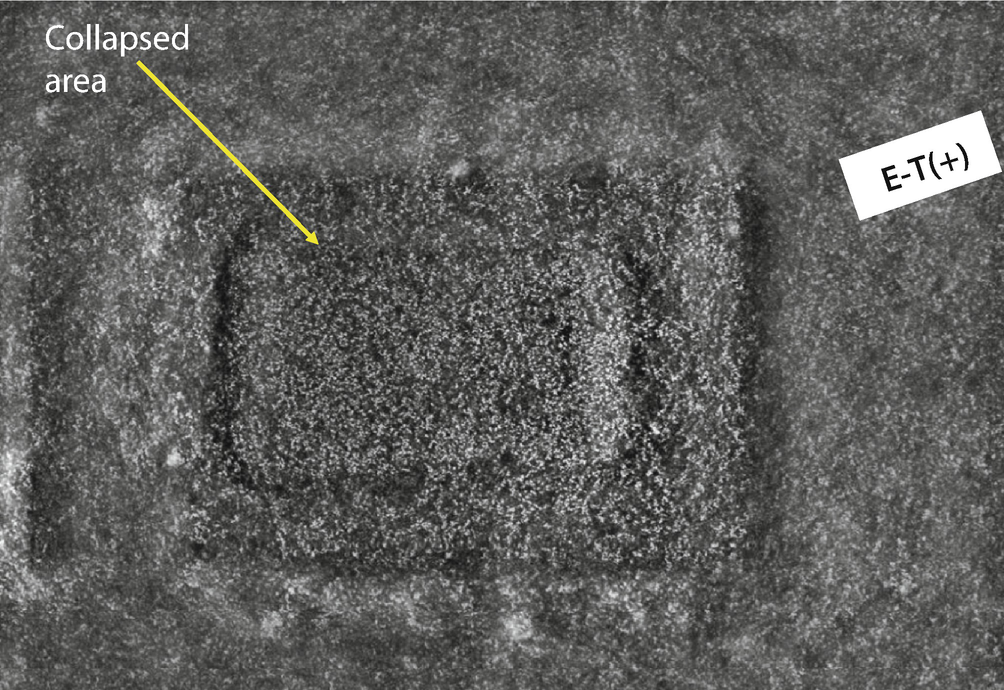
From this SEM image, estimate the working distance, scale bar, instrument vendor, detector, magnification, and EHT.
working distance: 11 mm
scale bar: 40000 nm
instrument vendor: Tescan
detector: SE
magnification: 0.5 K X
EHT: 20 kV